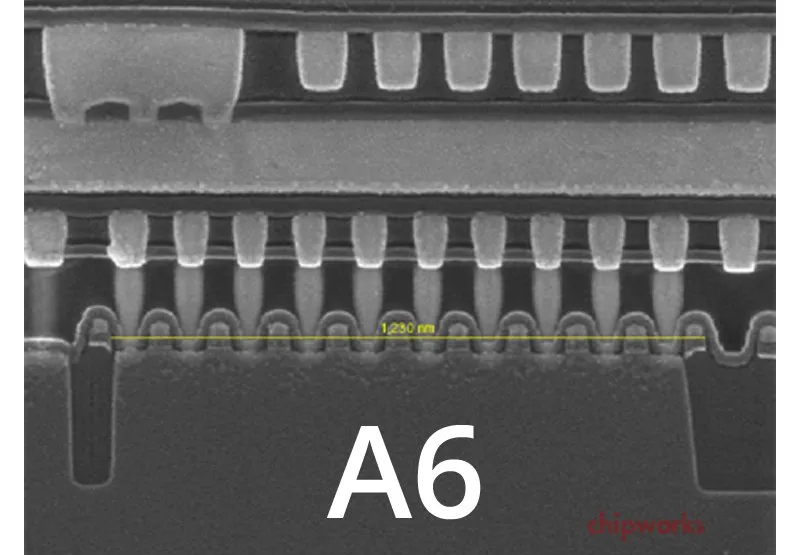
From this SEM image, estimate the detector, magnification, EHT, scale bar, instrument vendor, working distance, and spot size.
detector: TLD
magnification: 80 K X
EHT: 10 kV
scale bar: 200 nm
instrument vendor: FEI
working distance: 5.1 mm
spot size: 3